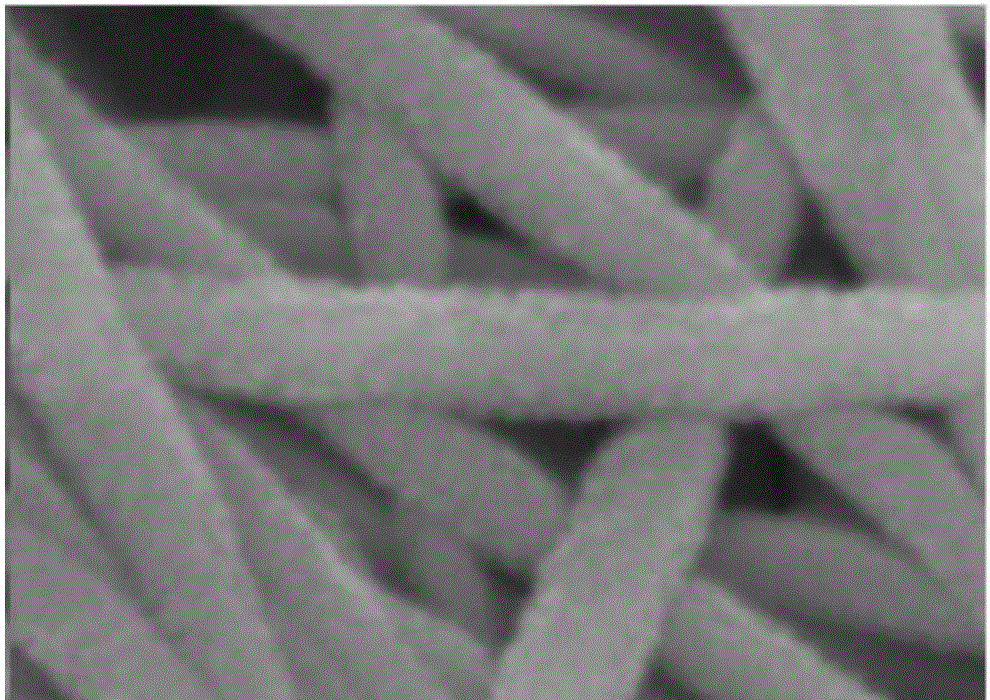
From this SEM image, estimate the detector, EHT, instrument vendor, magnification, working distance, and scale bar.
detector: SE(U)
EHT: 5 kV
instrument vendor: Hitachi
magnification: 250 K X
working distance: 8.9 mm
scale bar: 200 nm